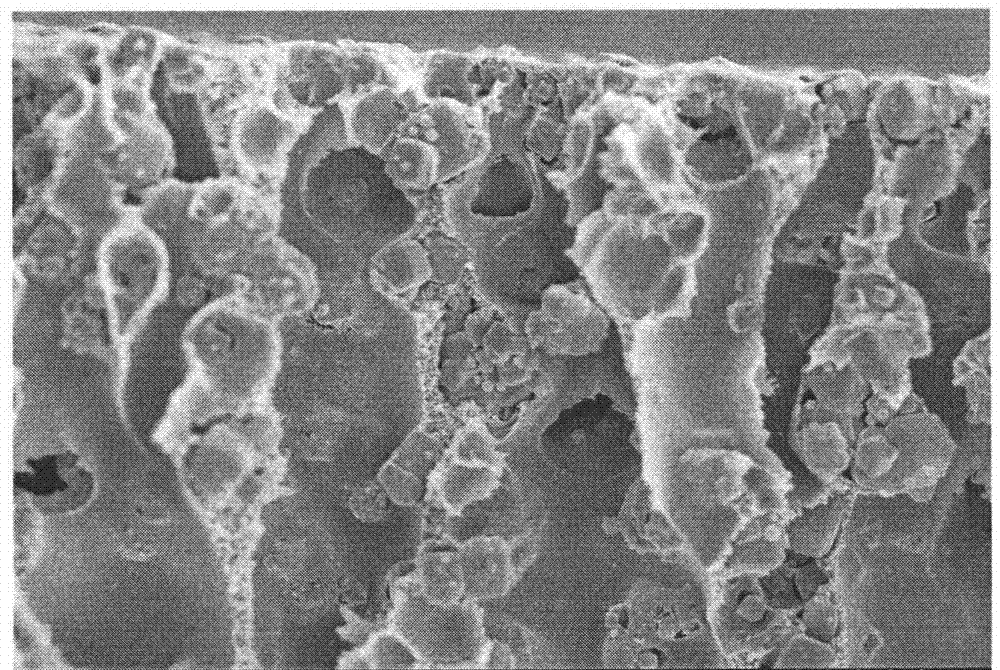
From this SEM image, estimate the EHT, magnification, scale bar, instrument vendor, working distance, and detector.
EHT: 5 kV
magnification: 2 K X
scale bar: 20000 nm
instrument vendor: Hitachi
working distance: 7.1 mm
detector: SE(M)